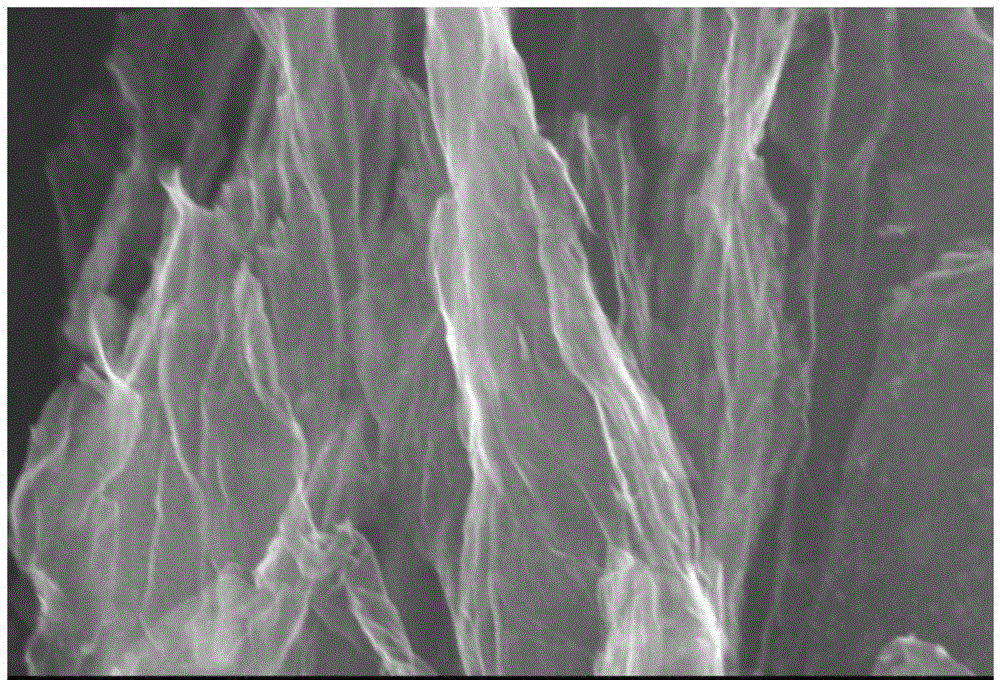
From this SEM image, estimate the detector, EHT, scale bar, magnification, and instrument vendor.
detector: SEI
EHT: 20 kV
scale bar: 1000 nm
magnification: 20 K X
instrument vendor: JEOL